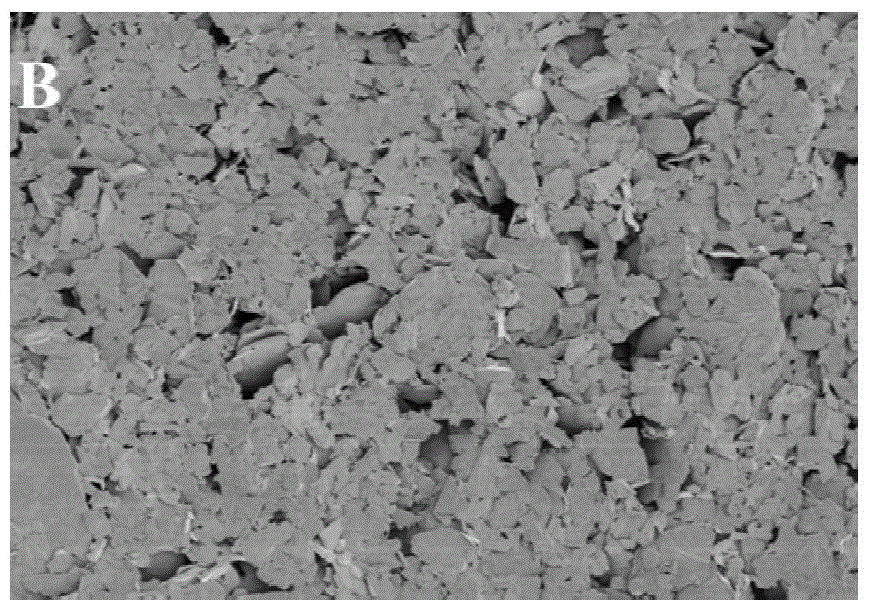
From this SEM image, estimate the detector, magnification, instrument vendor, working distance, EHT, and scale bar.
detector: SE(UL)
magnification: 0.5 K X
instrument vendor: Hitachi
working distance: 9.1 mm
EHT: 1 kV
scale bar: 100000 nm